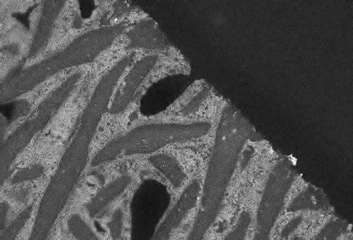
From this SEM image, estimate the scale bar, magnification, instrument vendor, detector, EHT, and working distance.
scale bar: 100000 nm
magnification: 0.35 K X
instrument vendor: Hitachi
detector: MD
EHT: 5 kV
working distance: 8.6 mm